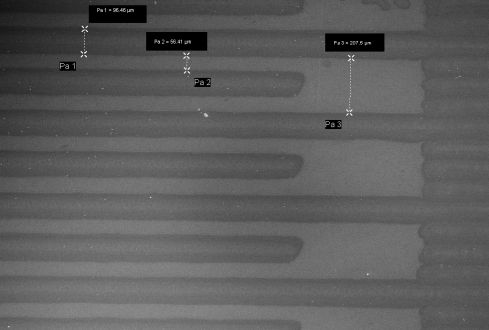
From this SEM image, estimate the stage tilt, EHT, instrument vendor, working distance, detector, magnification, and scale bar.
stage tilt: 0°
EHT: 5 kV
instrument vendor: Zeiss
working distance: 6.1 mm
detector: InLens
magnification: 0.146 K X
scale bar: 200000 nm